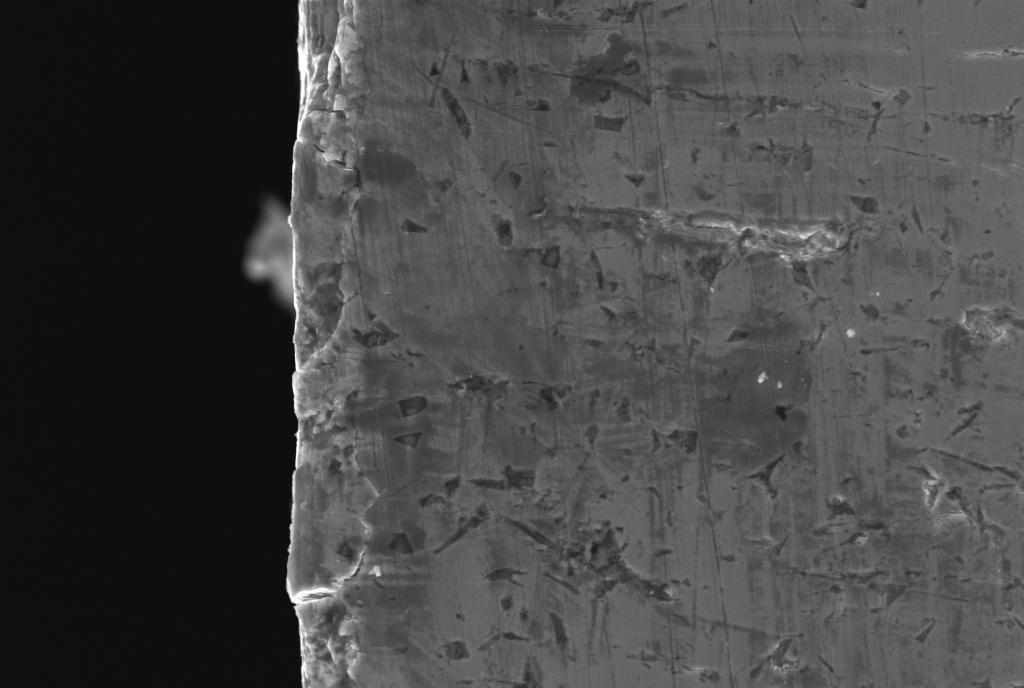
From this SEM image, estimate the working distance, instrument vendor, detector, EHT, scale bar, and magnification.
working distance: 30 mm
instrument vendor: Zeiss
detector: SE1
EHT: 20 kV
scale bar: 10000 nm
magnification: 2 K X